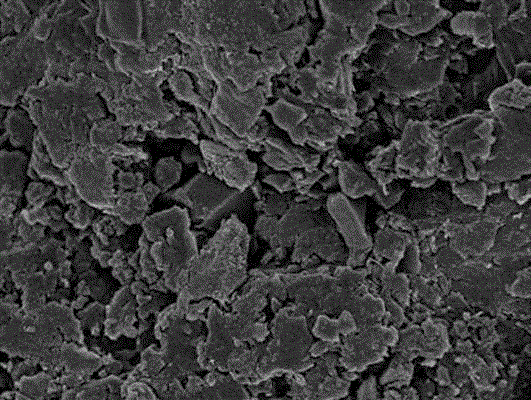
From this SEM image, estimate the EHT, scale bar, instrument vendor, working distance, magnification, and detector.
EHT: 3 kV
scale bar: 5000 nm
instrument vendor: Hitachi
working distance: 6.6 mm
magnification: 8 K X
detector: SE(M)